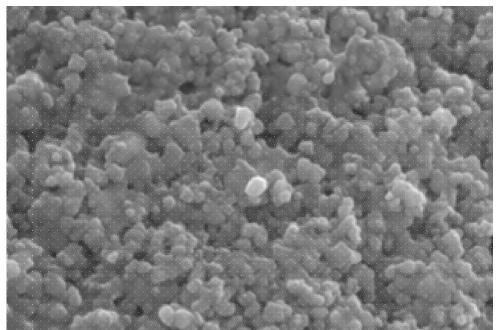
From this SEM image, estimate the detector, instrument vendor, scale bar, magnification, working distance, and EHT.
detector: SED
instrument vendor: JEOL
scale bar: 500 nm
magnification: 30 K X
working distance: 8 mm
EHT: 20 kV